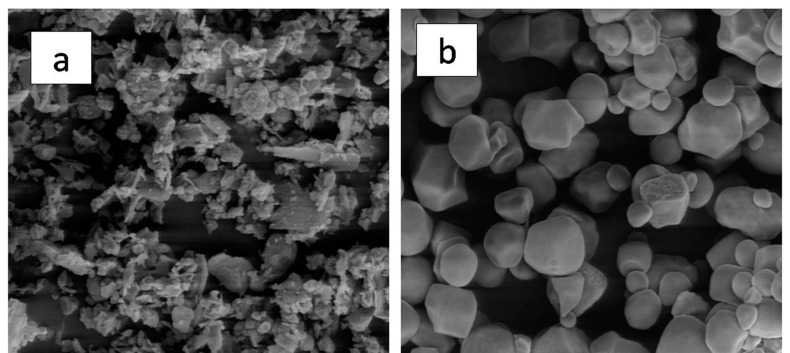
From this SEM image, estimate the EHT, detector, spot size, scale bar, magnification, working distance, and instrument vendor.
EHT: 10 kV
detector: LFD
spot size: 3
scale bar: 50000 nm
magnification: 3 K X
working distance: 10.1 mm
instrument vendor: FEI